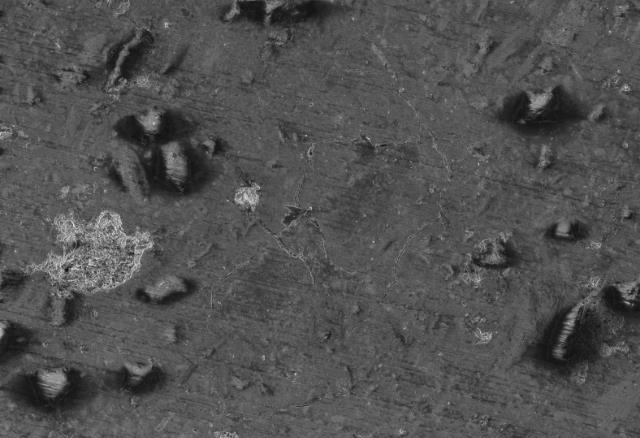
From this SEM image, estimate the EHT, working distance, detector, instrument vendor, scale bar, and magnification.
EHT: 4 kV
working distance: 5.9 mm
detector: InLens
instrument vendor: Zeiss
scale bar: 20000 nm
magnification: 1 K X